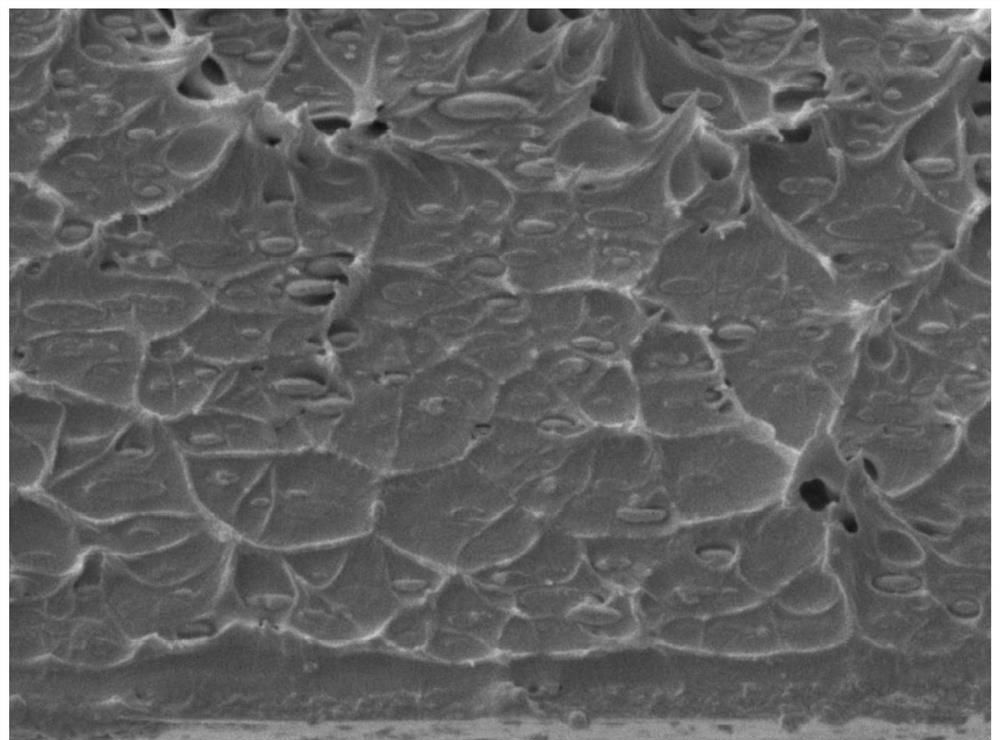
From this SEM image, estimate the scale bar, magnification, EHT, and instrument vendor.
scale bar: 1000 nm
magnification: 4 K X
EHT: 5 kV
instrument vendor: JEOL